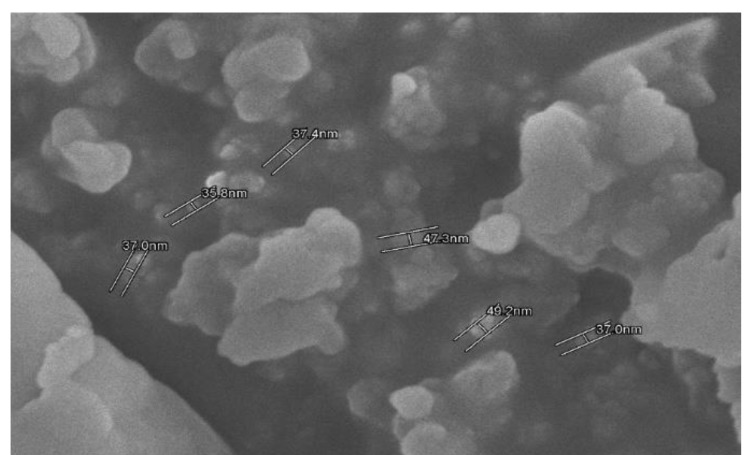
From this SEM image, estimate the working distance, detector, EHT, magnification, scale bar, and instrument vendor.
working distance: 9.2 mm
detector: SE(M)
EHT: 5 kV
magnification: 60 K X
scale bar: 500 nm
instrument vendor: Hitachi